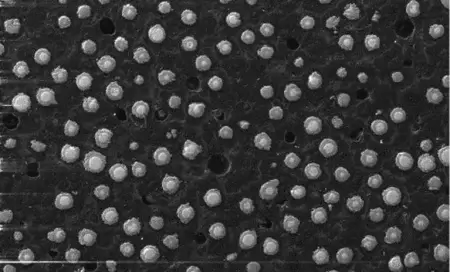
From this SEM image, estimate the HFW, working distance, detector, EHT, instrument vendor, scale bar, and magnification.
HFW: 700 µm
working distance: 15 mm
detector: SE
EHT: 20 kV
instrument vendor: Tescan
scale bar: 100000 nm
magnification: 0.2 K X